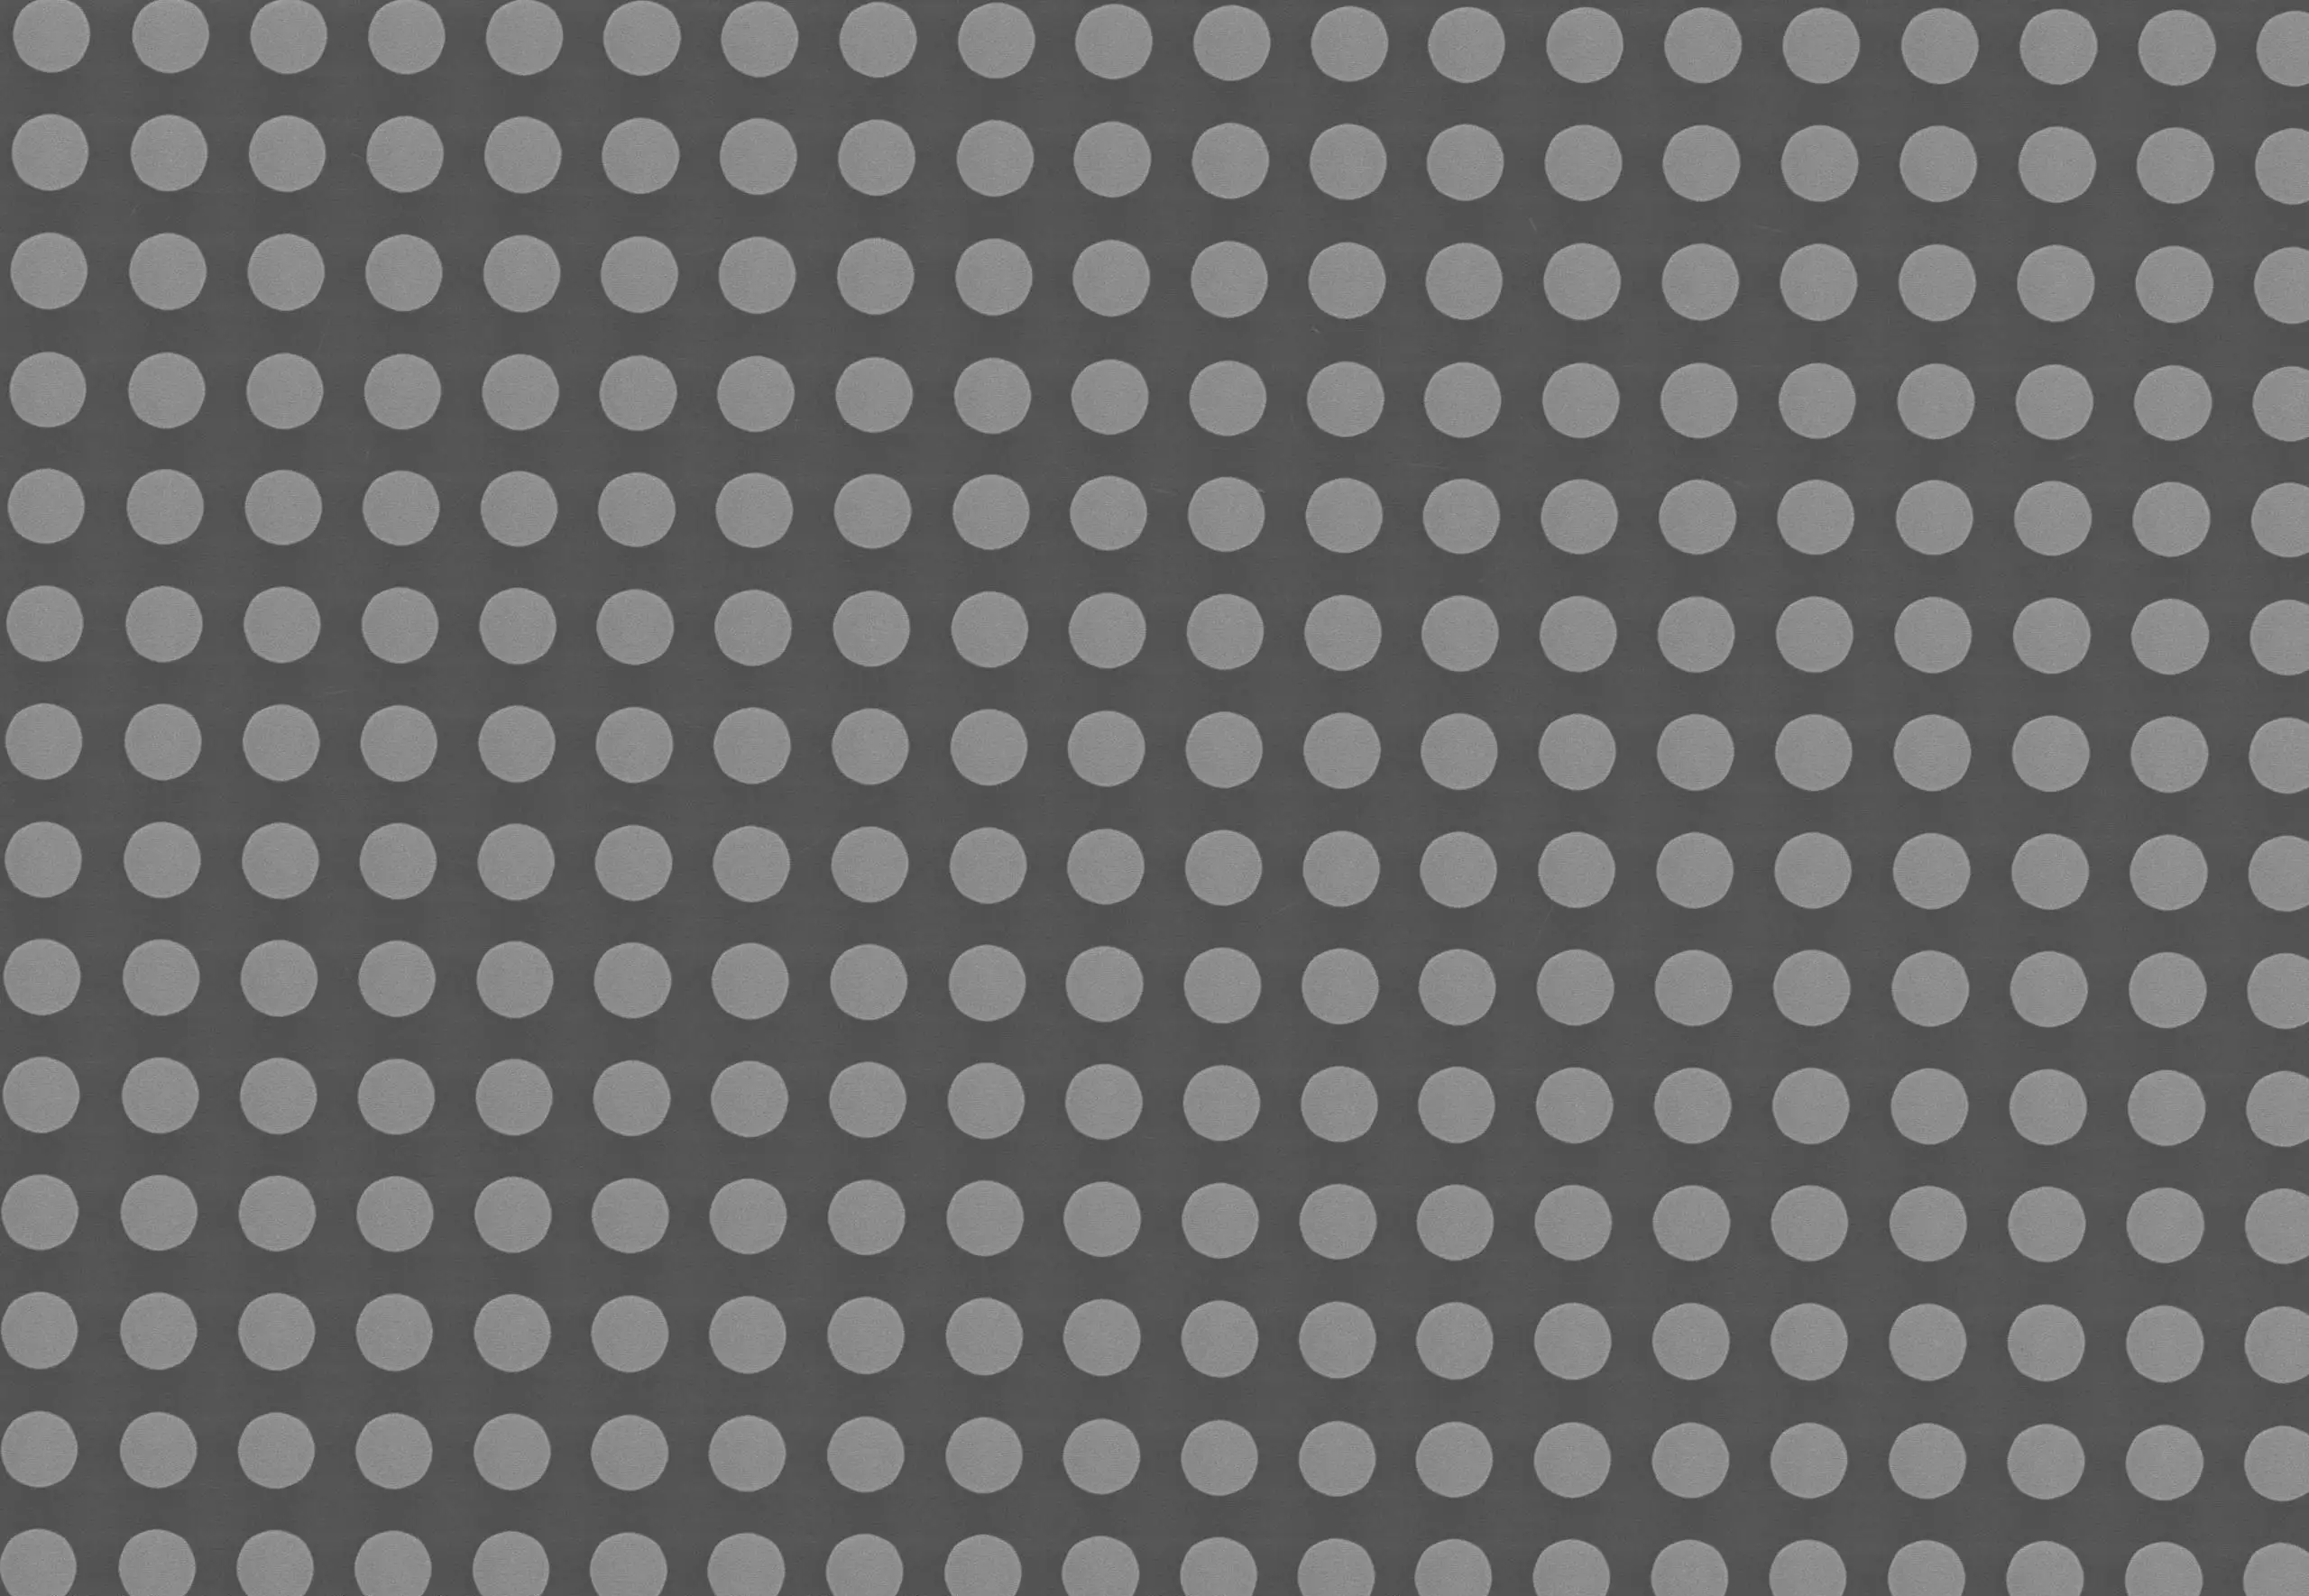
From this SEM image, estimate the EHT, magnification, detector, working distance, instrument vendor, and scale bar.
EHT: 15 kV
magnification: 1.1 K X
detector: SED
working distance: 26 mm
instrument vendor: JEOL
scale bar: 10000 nm